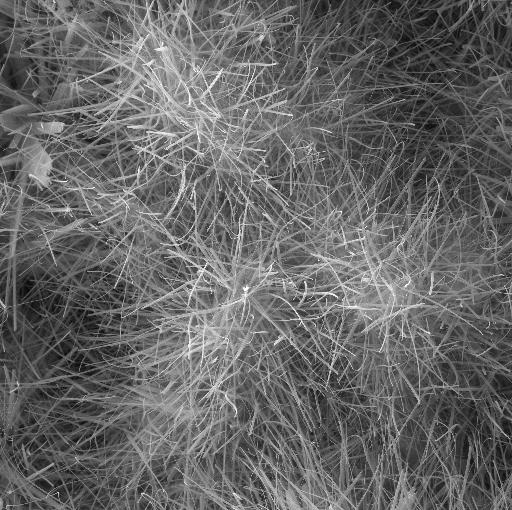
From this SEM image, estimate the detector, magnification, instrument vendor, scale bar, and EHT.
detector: SE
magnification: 5 K X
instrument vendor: Tescan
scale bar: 5000 nm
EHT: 20 kV